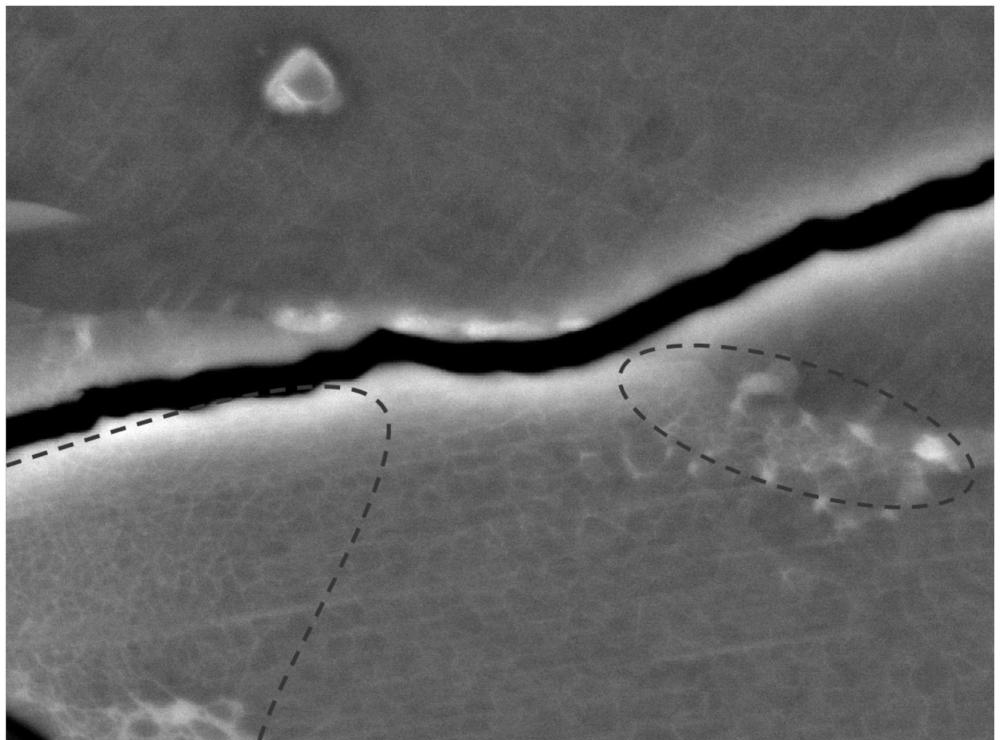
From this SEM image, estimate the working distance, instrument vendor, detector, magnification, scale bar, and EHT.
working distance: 3.3 mm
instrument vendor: JEOL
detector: BED-C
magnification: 20 K X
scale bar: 1000 nm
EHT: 15 kV